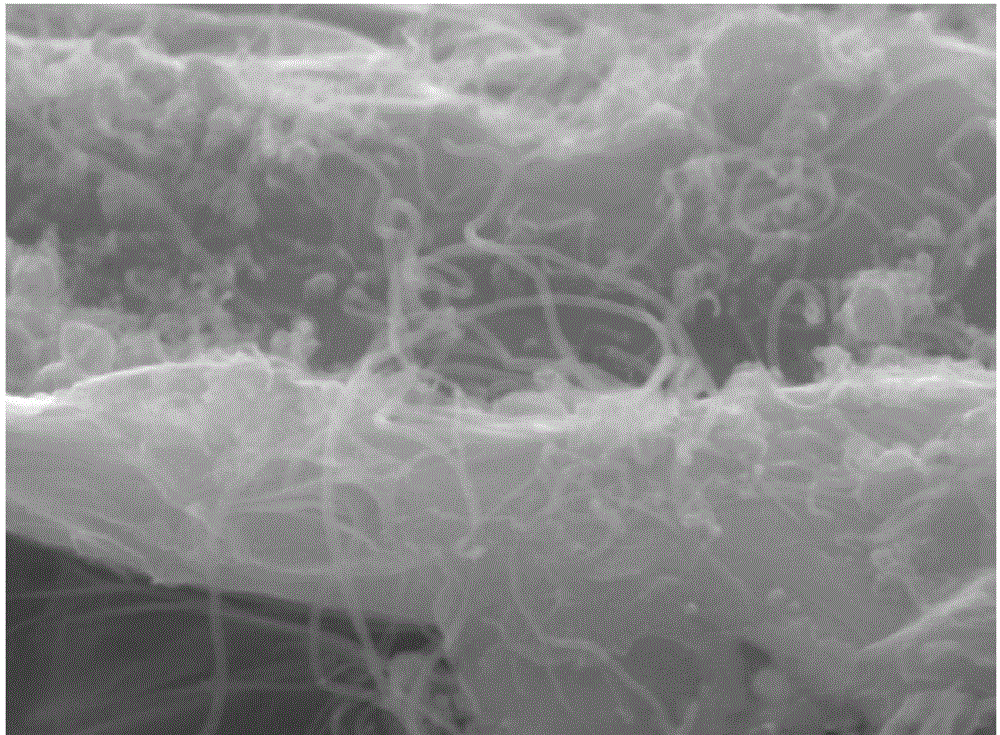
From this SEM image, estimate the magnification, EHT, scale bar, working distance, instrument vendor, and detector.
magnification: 60 K X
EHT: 10 kV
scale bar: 100 nm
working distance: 9.9 mm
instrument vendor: JEOL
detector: SEI